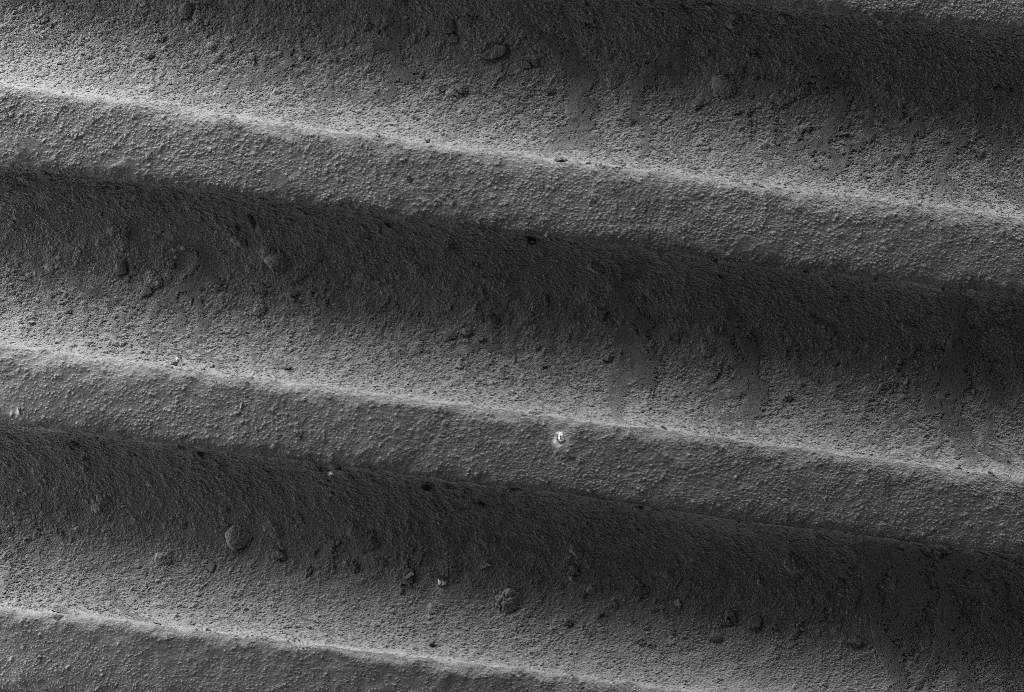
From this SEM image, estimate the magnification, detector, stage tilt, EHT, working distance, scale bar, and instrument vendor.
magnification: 0.096 K X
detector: SE2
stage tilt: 0°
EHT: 5 kV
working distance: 7.6 mm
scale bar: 200000 nm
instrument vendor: Zeiss